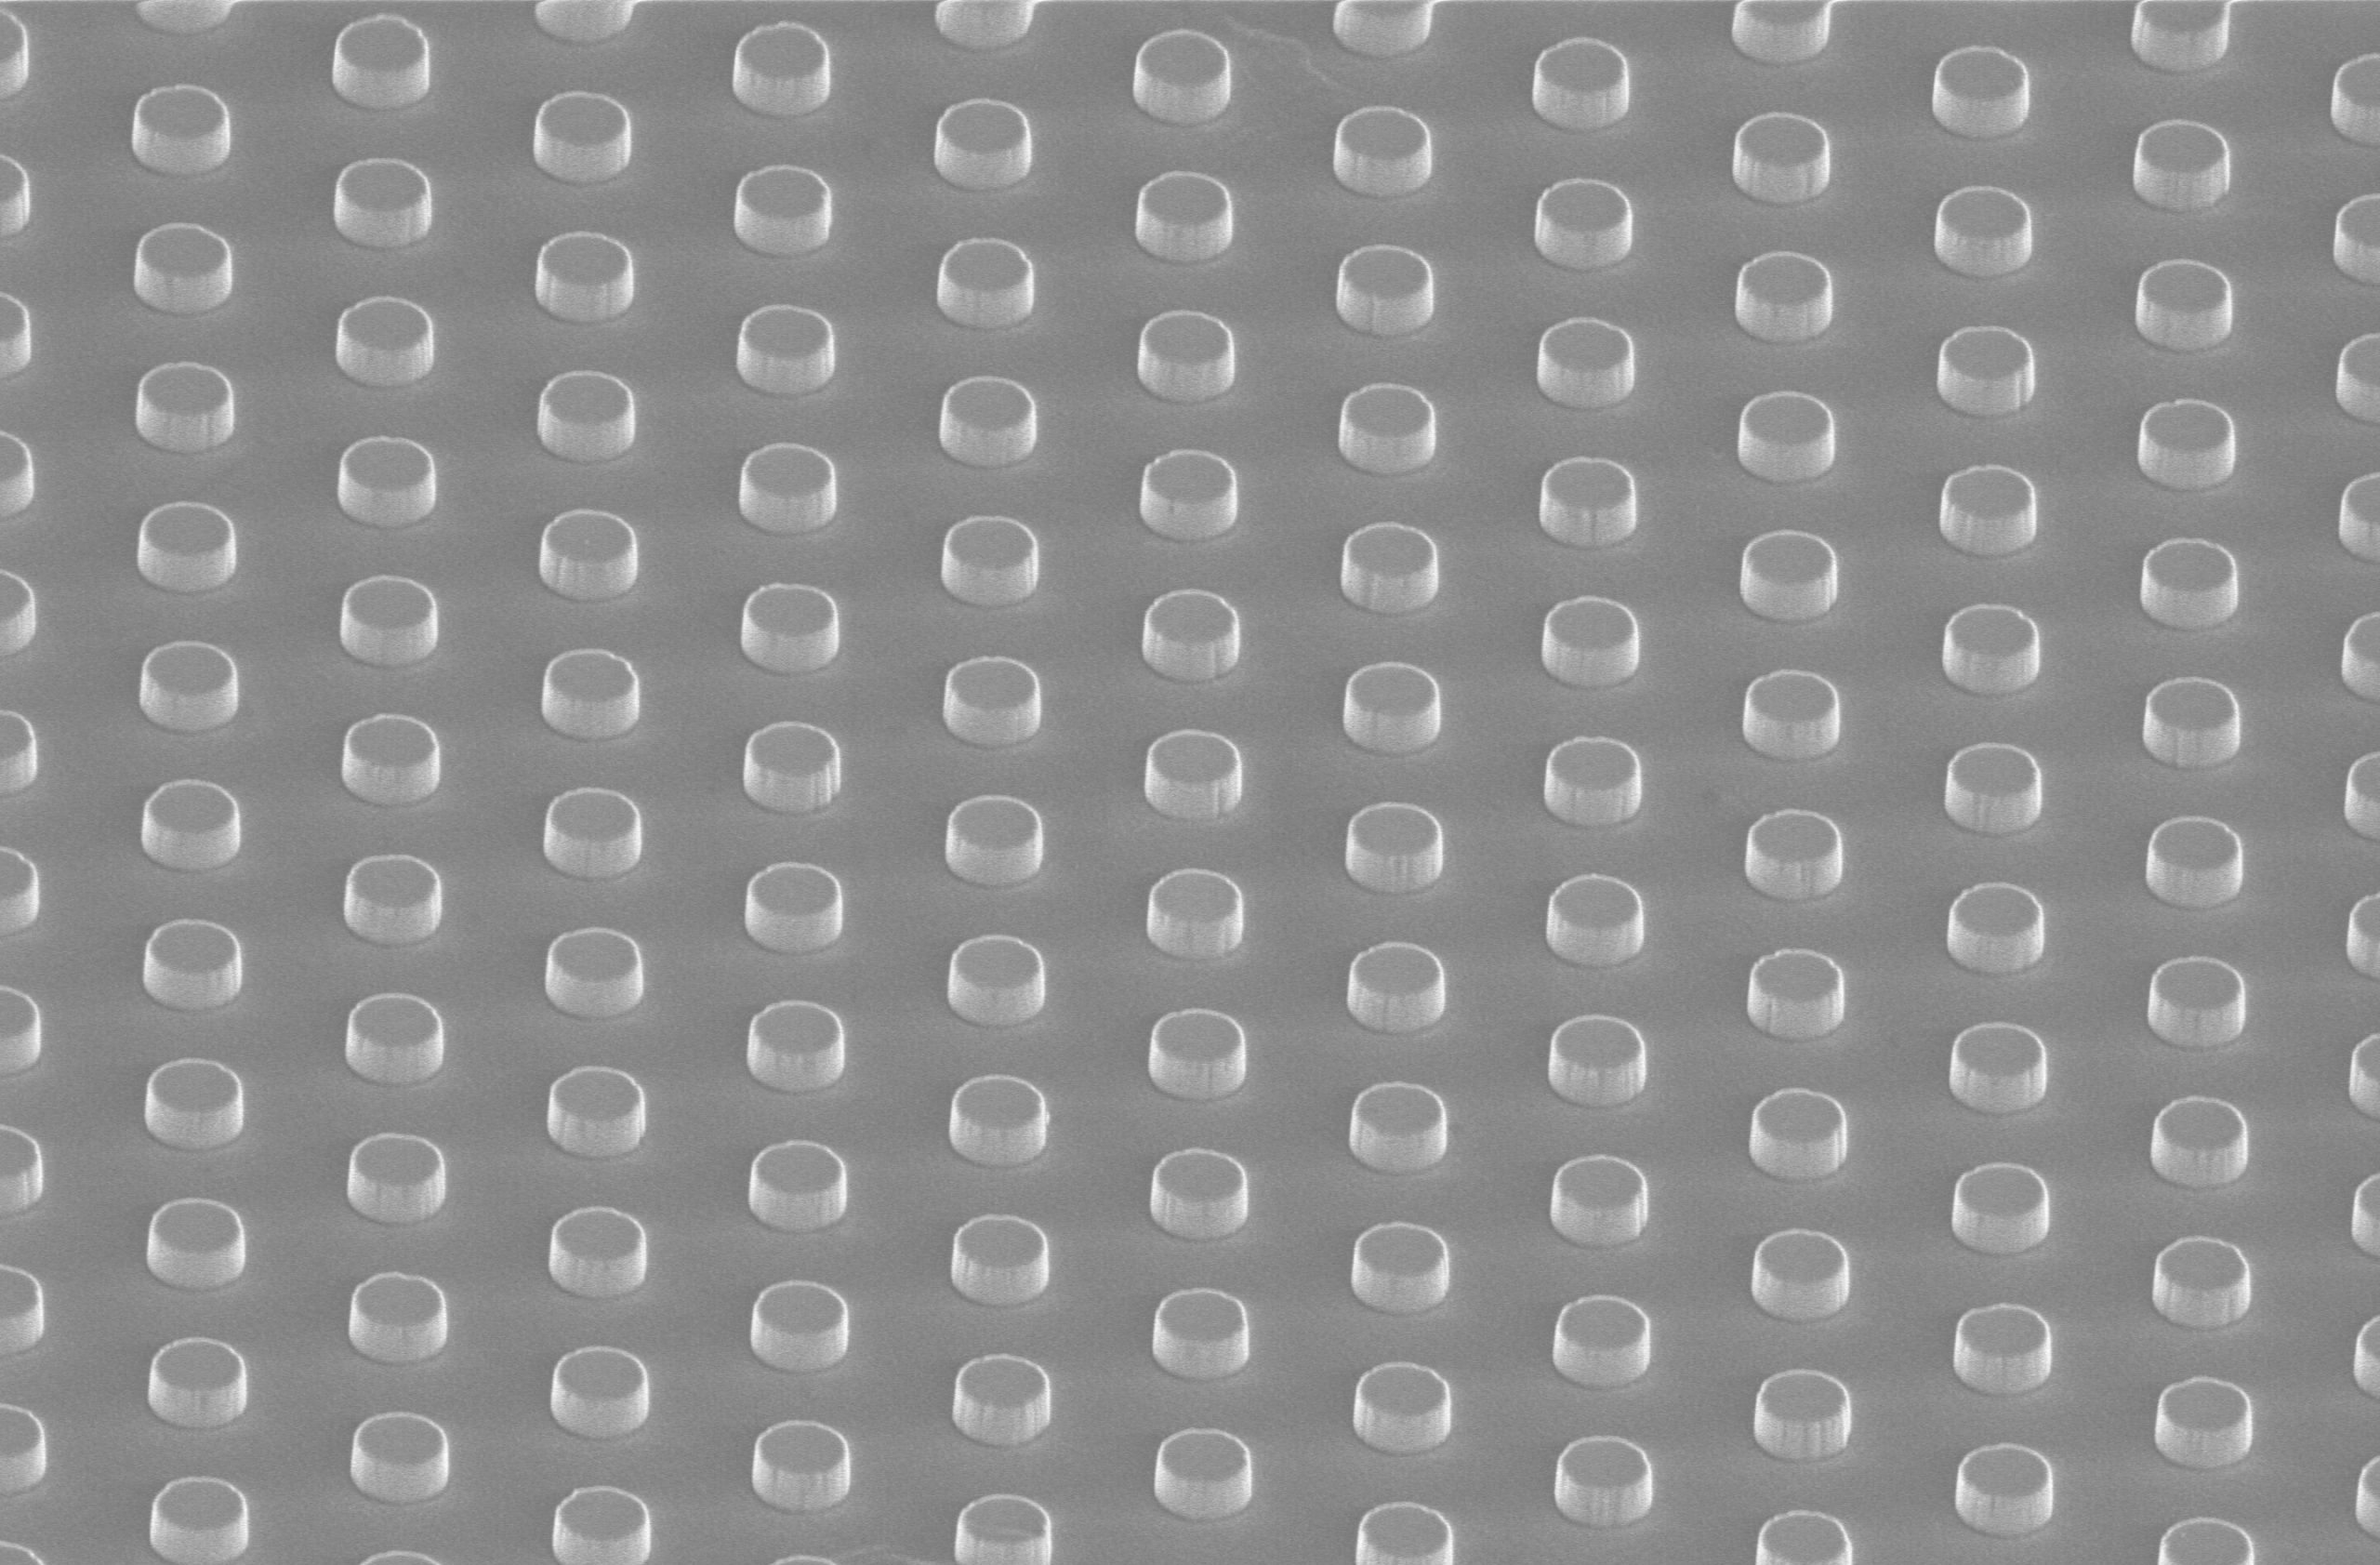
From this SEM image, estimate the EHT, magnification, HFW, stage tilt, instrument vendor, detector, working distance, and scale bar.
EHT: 2 kV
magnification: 10 K X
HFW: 20.7 µm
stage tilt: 52°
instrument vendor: FEI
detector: TLD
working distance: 3.8 mm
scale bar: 5000 nm